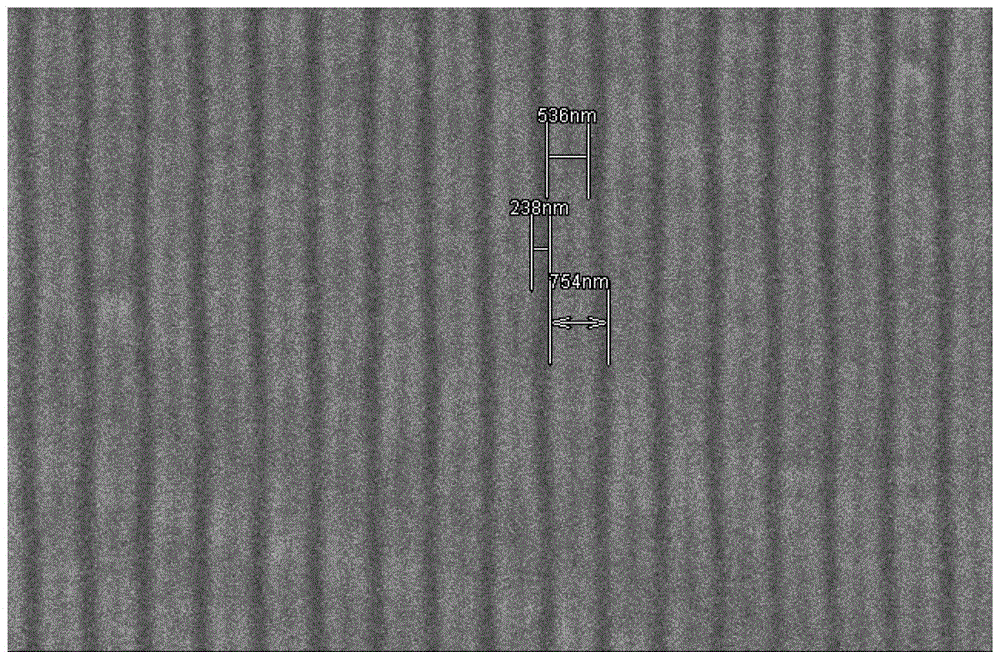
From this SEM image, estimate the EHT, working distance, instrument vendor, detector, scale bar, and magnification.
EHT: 1.5 kV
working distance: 8.7 mm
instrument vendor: Hitachi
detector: SE(L)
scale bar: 5000 nm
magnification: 10 K X